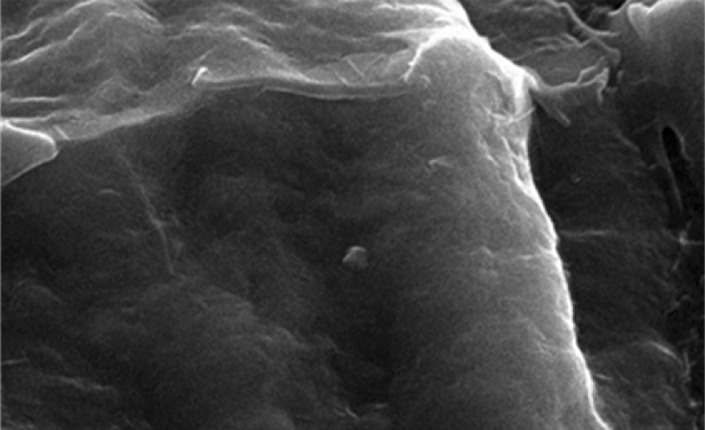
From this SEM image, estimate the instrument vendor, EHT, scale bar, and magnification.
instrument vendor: JEOL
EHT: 20 kV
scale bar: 2000 nm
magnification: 6 K X